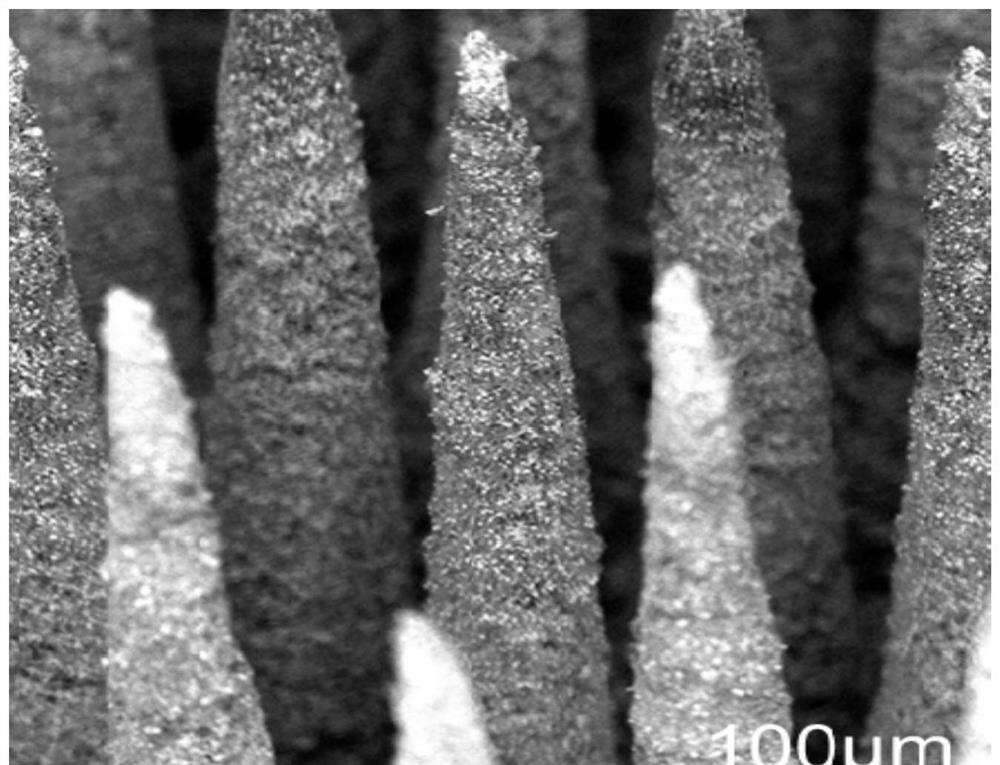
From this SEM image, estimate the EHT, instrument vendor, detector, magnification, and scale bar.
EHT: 10 kV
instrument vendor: other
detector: BSD Full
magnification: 0.23 K X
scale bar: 300000 nm